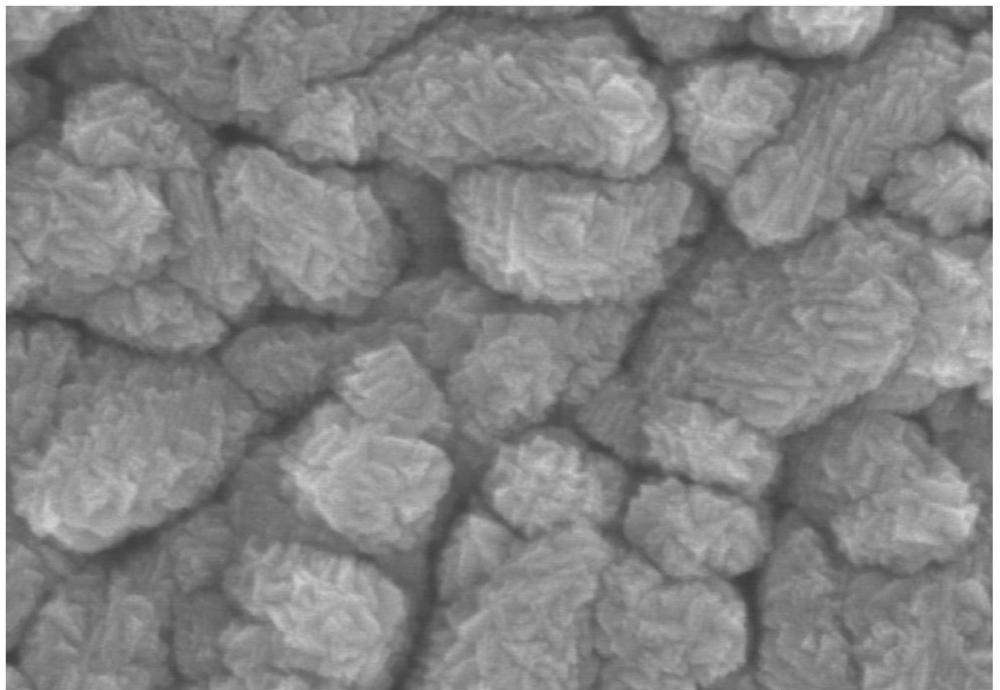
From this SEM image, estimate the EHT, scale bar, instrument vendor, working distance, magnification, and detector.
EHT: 5 kV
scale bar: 500 nm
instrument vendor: Hitachi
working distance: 9.5 mm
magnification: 100 K X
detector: SE(TUL)